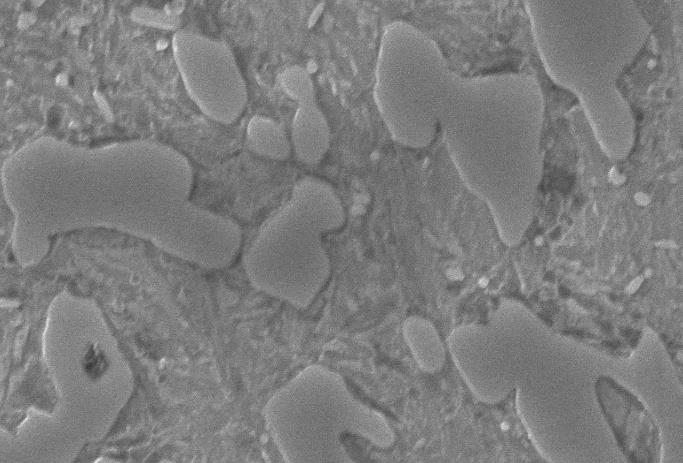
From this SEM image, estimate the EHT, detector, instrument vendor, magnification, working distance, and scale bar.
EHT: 20 kV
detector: SEI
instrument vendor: JEOL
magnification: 9 K X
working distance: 12 mm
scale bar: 2000 nm